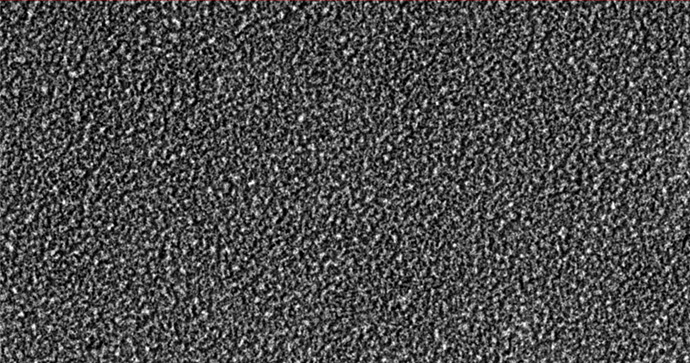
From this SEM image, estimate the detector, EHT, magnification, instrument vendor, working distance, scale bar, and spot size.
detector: SEI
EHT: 15 kV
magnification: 2 K X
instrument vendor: JEOL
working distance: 19 mm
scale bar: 10000 nm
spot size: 50